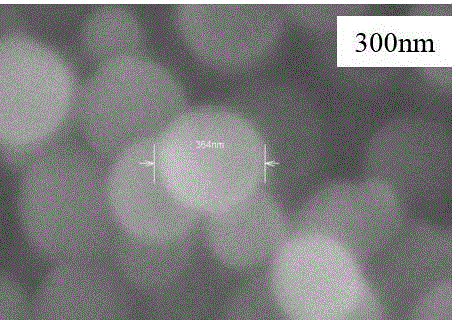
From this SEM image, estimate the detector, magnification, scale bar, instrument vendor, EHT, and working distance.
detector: SE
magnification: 85 K X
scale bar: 500 nm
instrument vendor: Hitachi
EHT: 15 kV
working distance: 13.6 mm